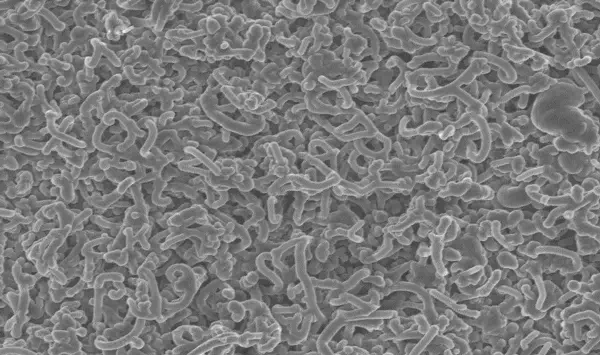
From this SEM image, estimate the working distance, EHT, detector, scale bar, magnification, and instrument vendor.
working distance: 9.3 mm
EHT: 5 kV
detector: InLens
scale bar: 1000 nm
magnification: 8.97 K X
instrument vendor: Zeiss